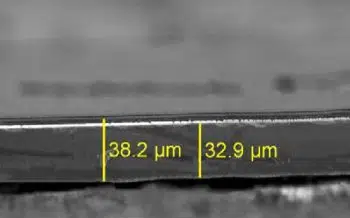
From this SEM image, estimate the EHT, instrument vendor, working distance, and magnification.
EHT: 5 kV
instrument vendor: Zeiss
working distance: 21 mm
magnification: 0.25 K X